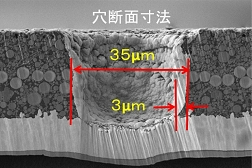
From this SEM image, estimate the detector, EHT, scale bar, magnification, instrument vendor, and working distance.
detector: SE(L,-150)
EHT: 5 kV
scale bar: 30000 nm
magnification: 1.5 K X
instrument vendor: Hitachi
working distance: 13.2 mm